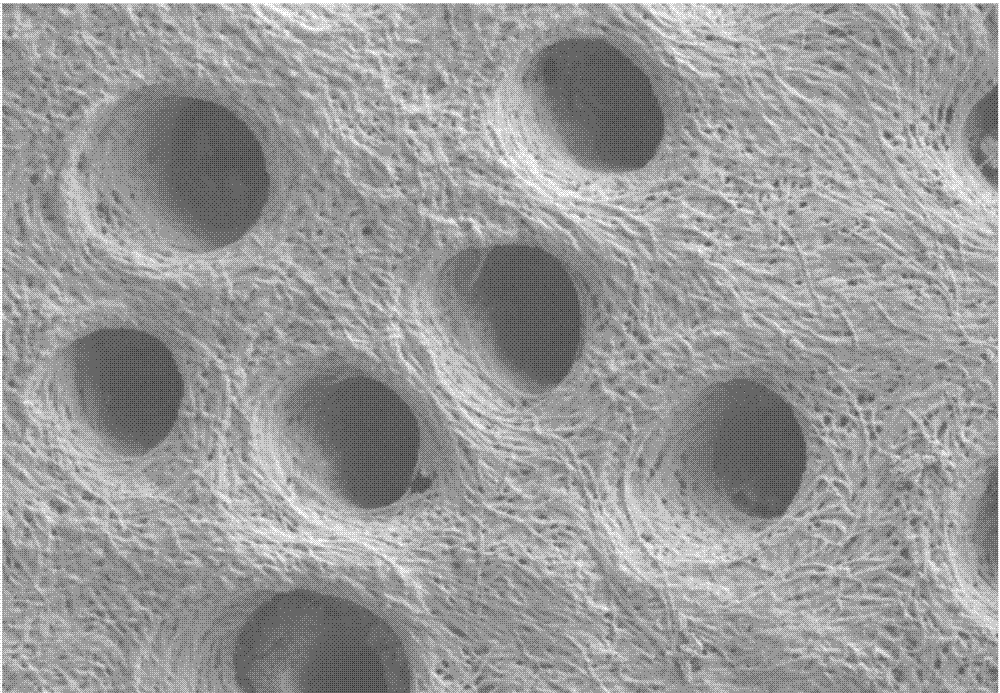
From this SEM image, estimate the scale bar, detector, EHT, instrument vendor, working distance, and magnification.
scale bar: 1000 nm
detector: SE2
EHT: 3 kV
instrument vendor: Zeiss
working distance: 5.3 mm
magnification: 6 K X